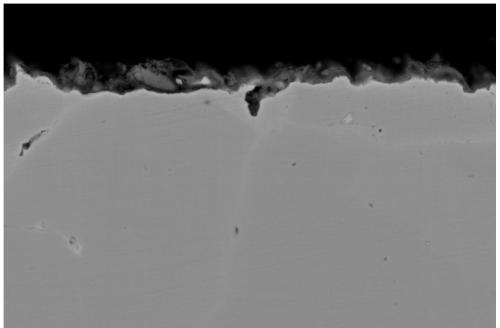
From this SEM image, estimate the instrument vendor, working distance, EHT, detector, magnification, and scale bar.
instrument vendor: Zeiss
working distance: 10.3 mm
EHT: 20 kV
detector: HDBSD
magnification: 2 K X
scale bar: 2000 nm